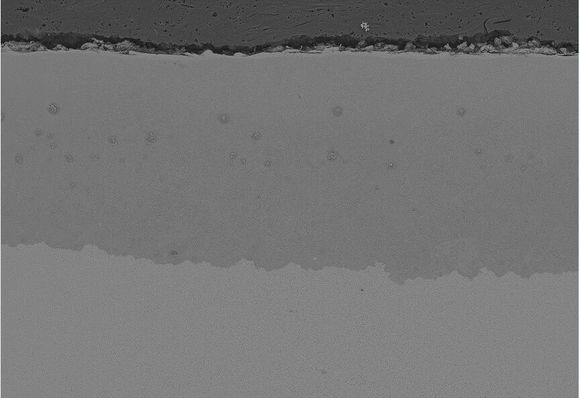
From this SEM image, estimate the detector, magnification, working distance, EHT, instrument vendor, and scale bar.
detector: BSE-COMP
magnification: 0.2 K X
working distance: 10.9 mm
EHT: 15 kV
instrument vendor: Hitachi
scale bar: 200000 nm